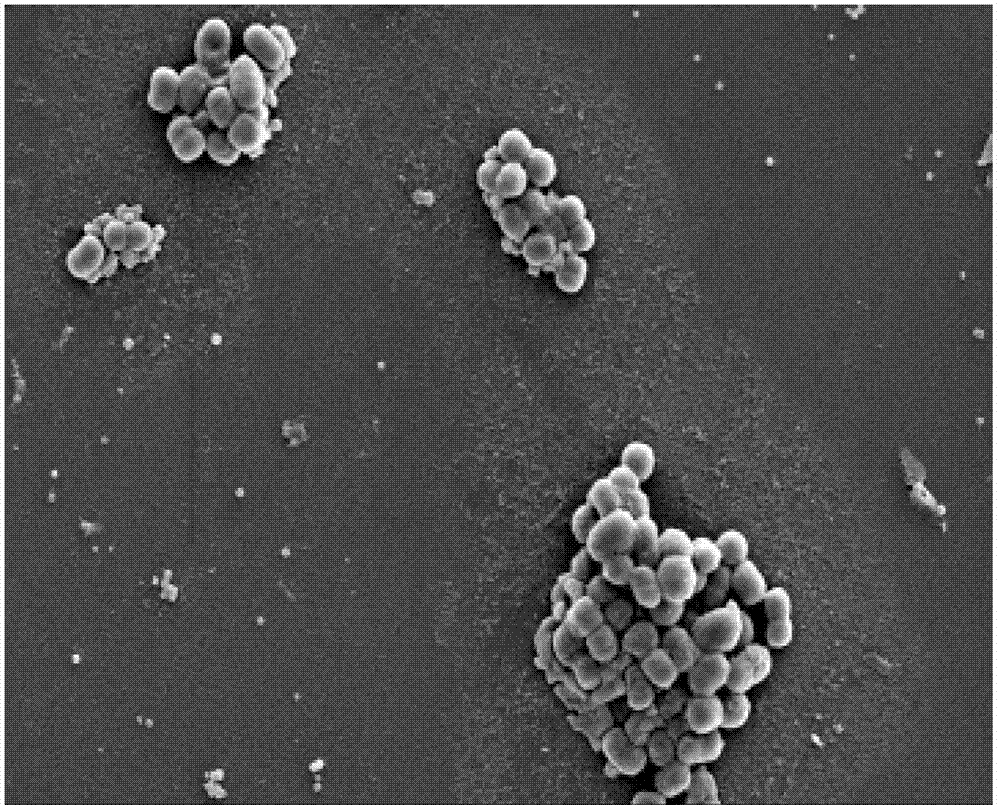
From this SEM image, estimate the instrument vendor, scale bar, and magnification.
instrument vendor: Hitachi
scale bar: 10000 nm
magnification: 5 K X